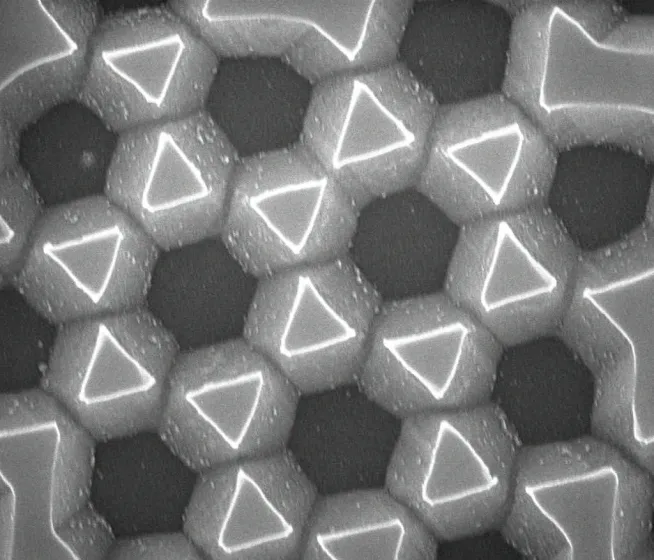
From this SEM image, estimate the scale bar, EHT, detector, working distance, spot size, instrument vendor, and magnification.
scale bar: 1000 nm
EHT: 10 kV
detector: TLD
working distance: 5.2 mm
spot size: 3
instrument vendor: FEI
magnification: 50 K X